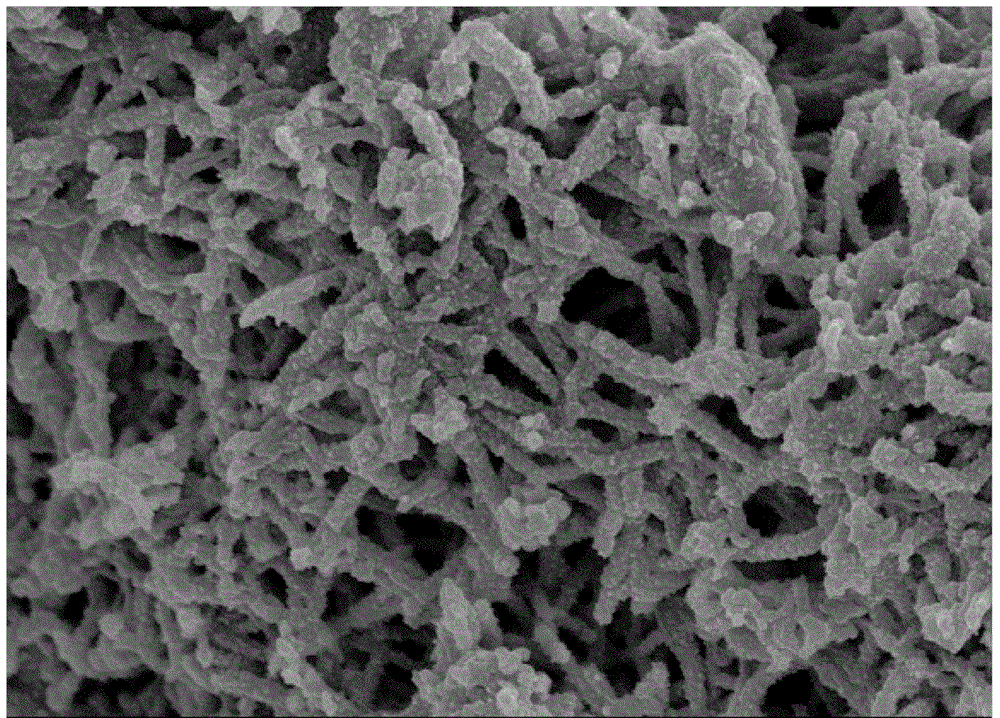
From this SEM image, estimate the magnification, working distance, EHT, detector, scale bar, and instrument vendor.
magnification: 30 K X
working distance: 8.6 mm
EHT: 3 kV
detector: SE(M)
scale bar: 1000 nm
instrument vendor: Hitachi